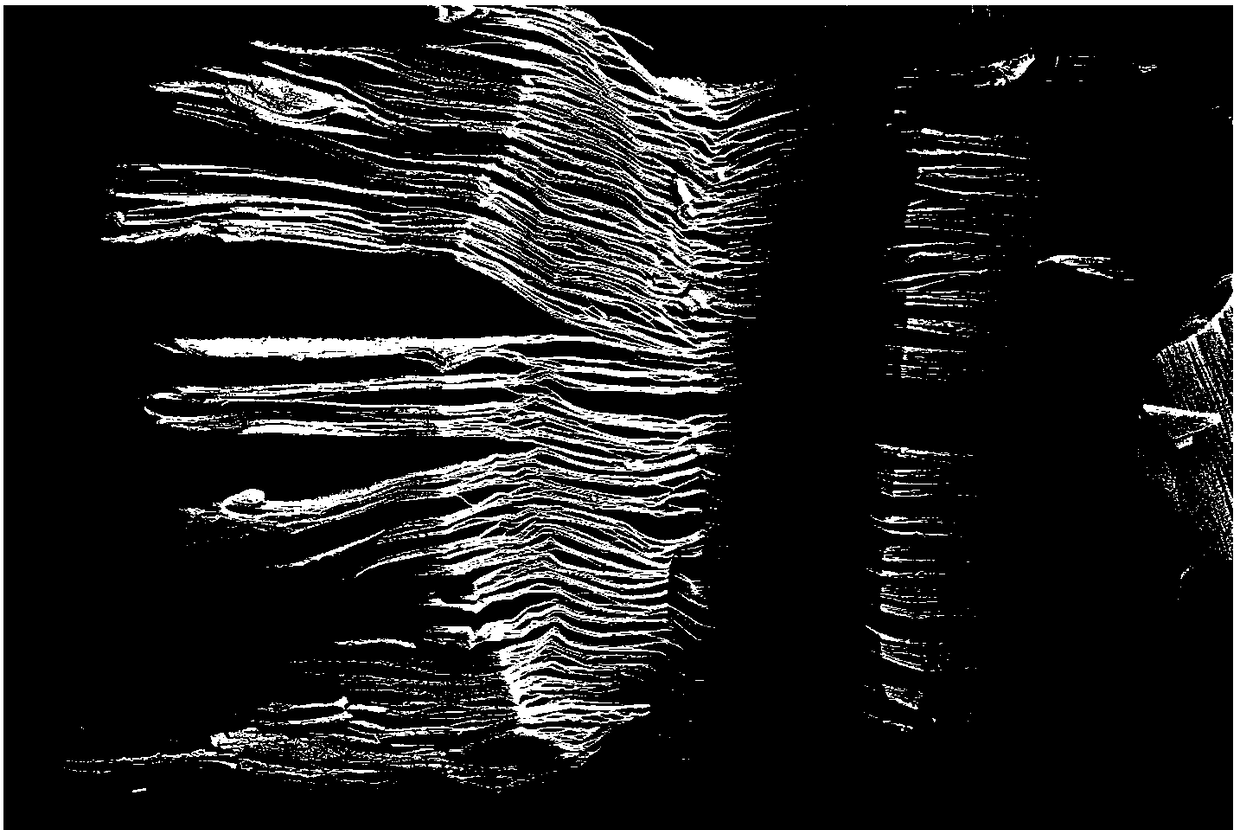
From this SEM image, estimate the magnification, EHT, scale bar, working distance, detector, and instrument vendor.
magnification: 5 K X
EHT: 5 kV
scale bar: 2000 nm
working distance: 5.7 mm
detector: SE2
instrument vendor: Zeiss